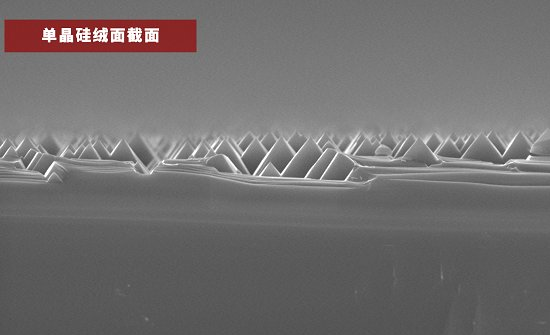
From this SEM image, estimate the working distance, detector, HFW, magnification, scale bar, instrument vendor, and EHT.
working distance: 7.385 mm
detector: SED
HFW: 25.4 µm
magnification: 5 K X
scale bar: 8000 nm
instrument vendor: other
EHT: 10 kV